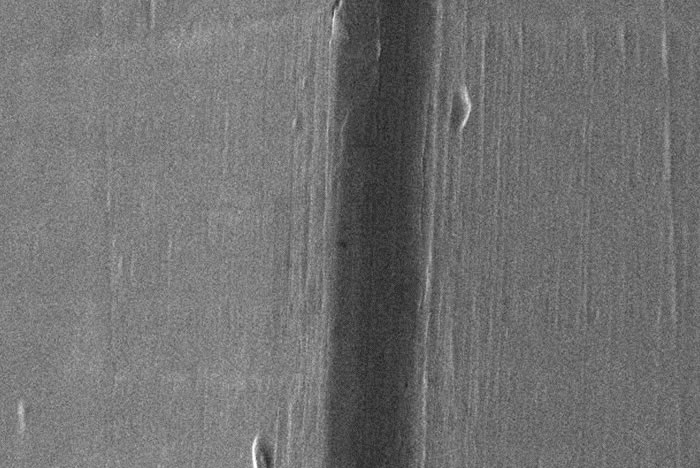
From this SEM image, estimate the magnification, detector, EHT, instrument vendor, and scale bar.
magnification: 1 K X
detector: SEI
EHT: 20 kV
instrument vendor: JEOL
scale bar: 10000 nm